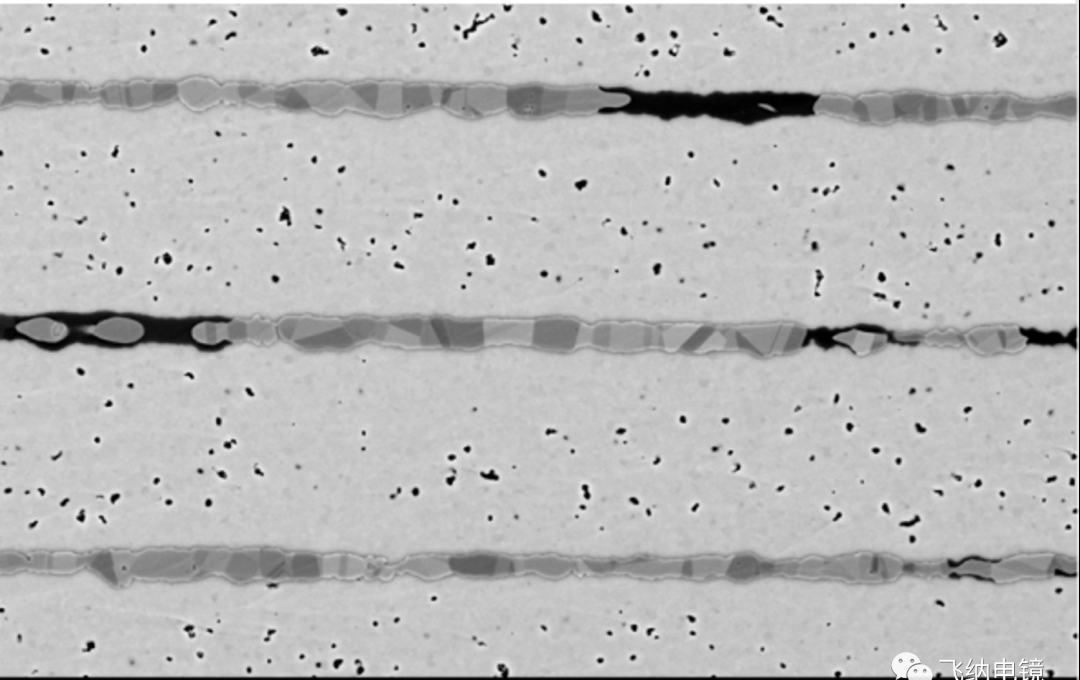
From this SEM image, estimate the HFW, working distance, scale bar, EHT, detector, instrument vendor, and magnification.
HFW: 51.9 µm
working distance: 10.568 mm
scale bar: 15000 nm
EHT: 10 kV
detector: BSD Full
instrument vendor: Thermo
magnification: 10 K X